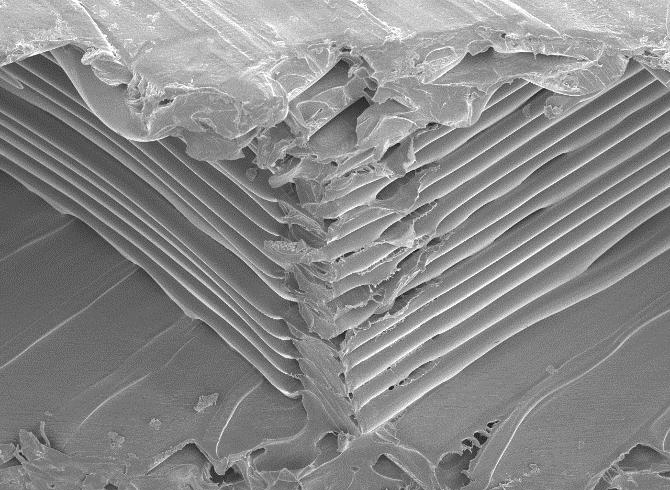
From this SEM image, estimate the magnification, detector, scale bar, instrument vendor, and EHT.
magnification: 0.05 K X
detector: SEI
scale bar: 100000 nm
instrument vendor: JEOL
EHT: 15 kV